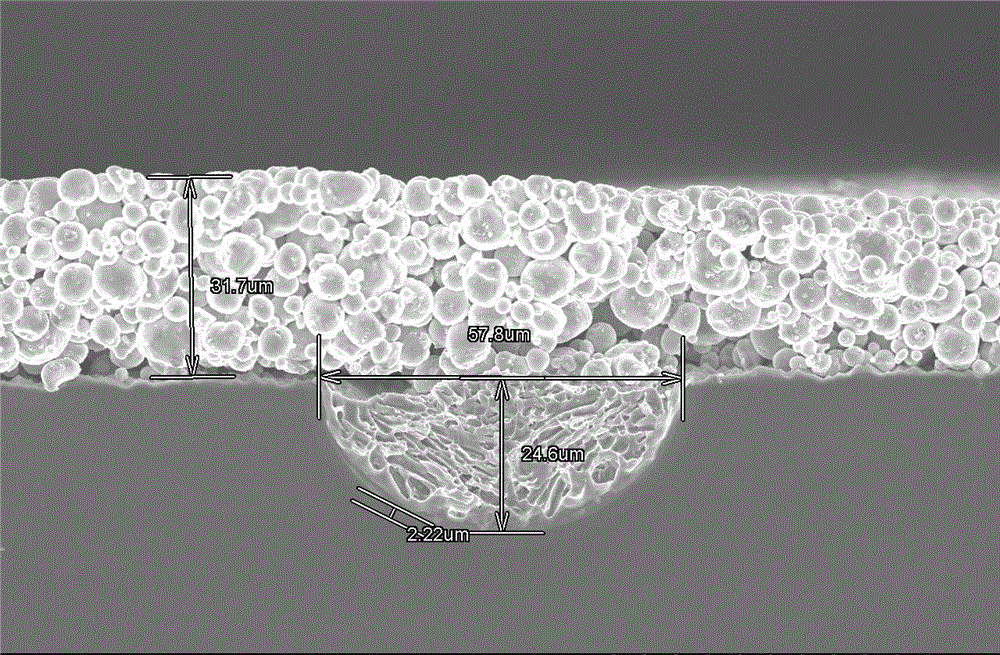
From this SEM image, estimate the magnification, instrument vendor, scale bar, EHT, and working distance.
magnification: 0.8 K X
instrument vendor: Hitachi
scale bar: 50000 nm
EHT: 10 kV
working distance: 8.5 mm